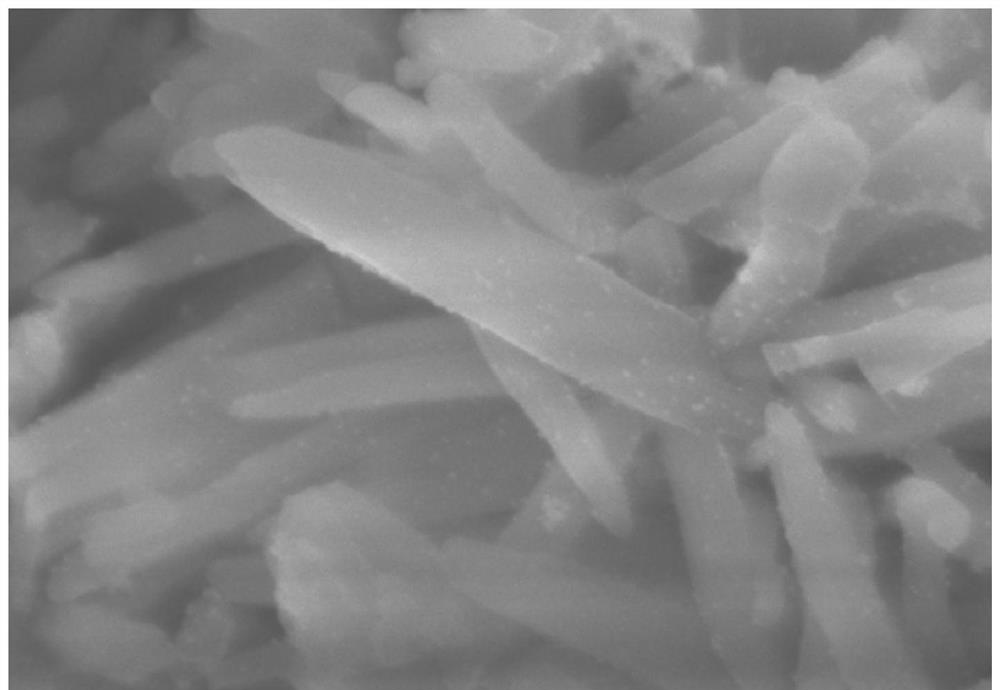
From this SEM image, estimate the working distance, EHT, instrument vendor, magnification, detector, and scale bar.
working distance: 4.5 mm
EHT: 3 kV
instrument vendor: Hitachi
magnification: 180 K X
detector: SE(UL)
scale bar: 300 nm